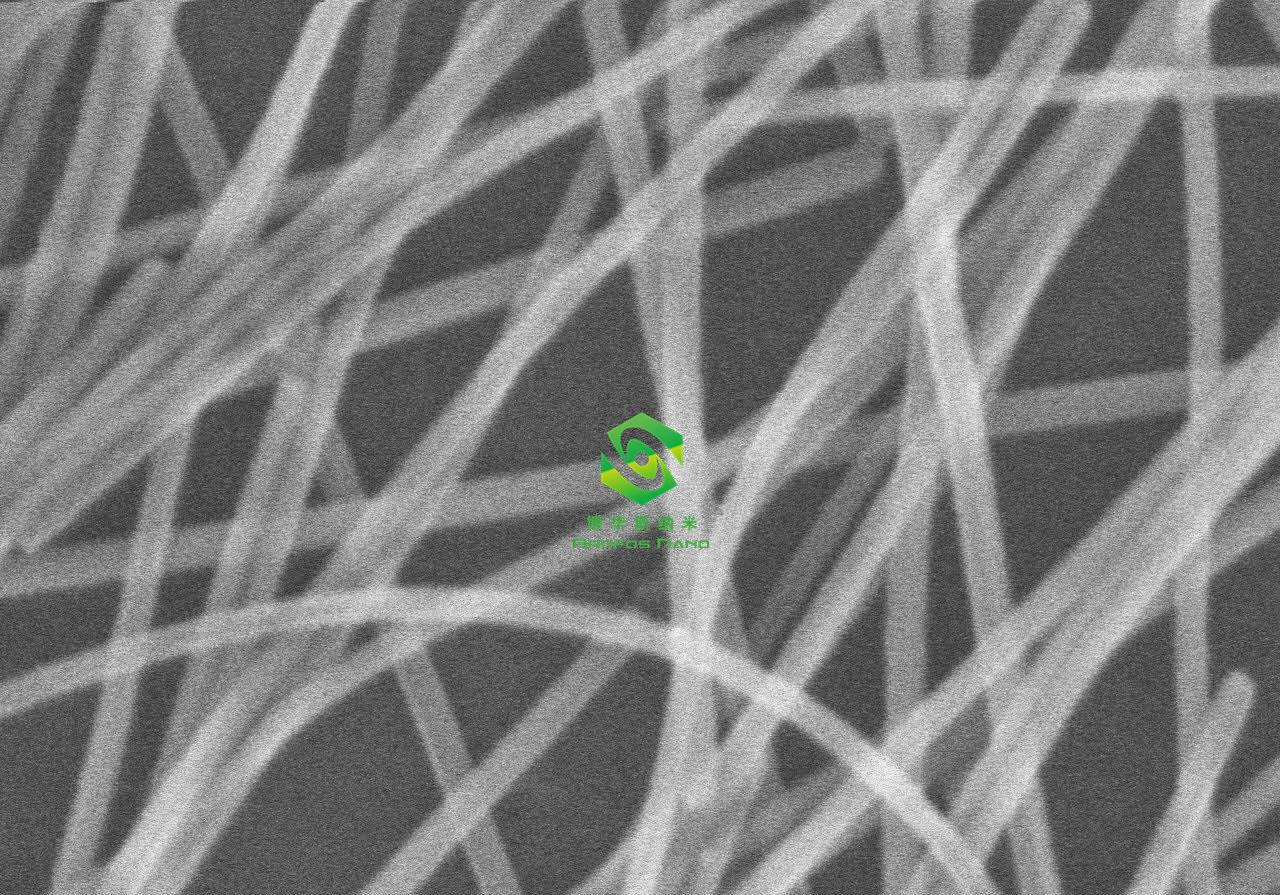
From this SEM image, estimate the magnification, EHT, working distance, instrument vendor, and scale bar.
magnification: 100 K X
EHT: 15 kV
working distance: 11.8 mm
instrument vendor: Hitachi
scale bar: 500 nm